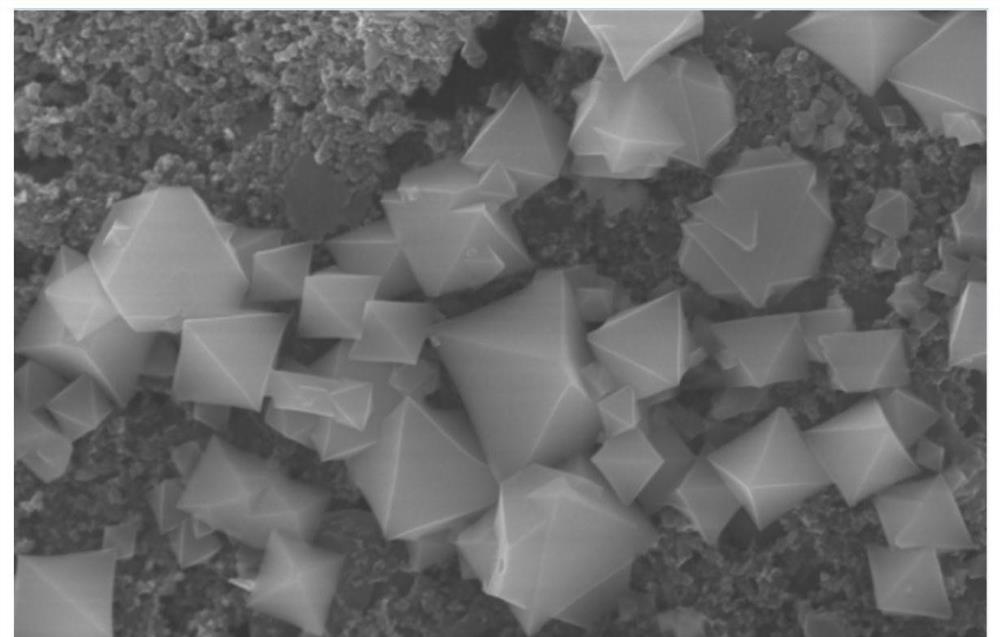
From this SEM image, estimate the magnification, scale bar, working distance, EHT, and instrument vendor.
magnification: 10 K X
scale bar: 5000 nm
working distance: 9.9 mm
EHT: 15 kV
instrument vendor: Hitachi